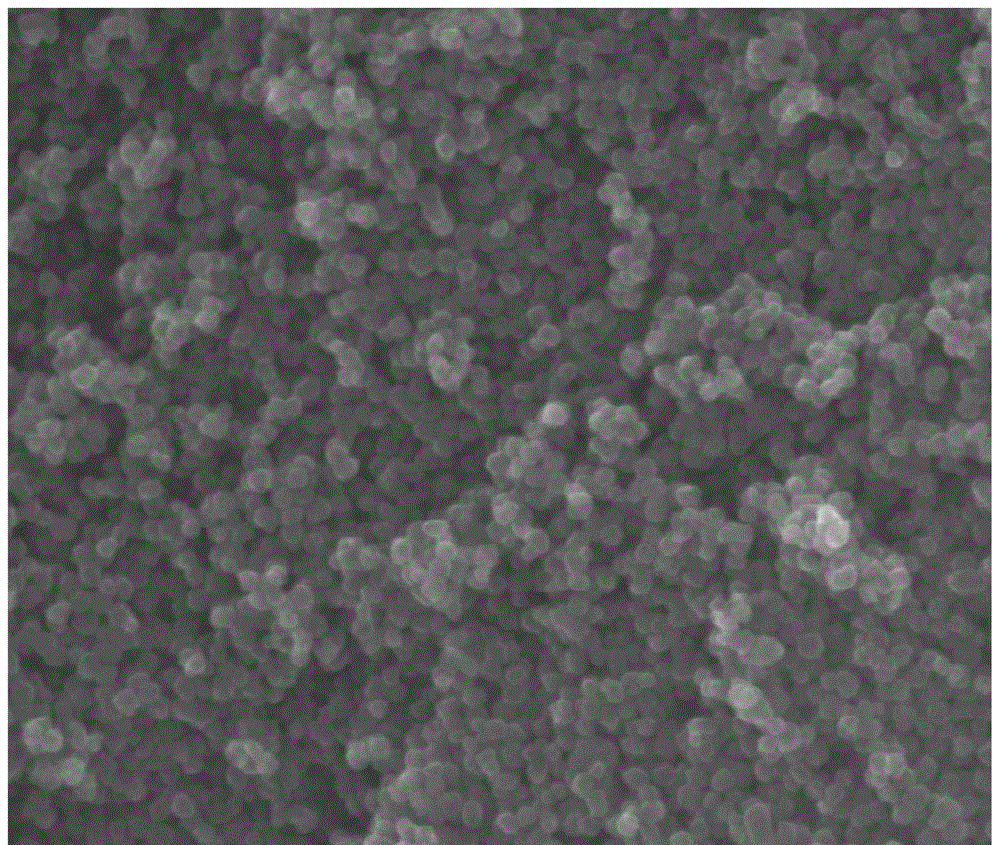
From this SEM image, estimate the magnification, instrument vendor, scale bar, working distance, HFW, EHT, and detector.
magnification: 60 K X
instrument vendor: FEI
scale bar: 1000 nm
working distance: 10 mm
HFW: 2.12 µm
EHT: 30 kV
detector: ETD